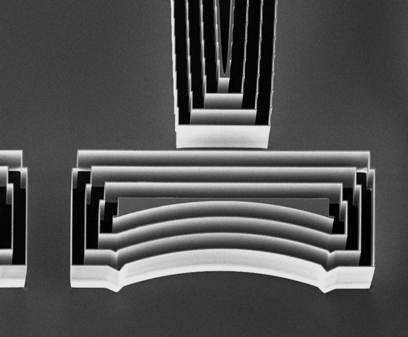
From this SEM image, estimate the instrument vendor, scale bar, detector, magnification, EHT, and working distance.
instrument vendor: Zeiss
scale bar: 30000 nm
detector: InLens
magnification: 0.697 K X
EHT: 5 kV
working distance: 3.2 mm